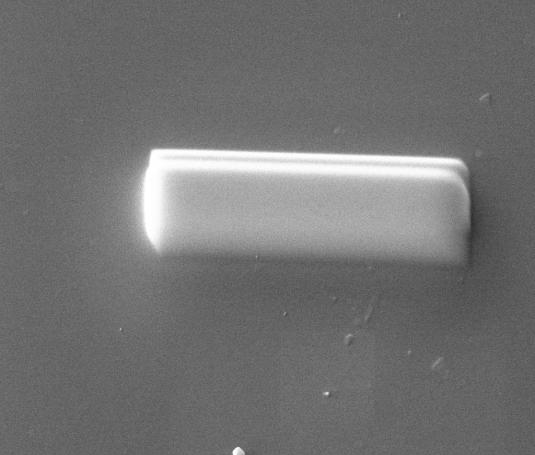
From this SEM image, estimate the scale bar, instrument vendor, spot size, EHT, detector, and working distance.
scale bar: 5000 nm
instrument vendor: FEI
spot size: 4.5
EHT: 20 kV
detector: CDEM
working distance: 10 mm